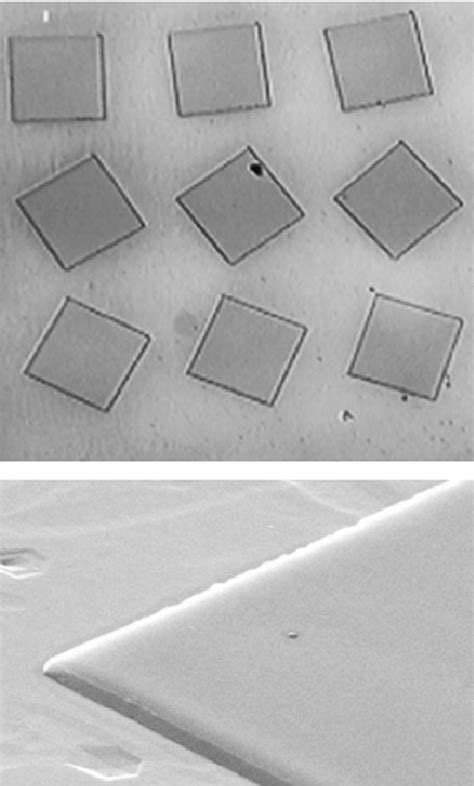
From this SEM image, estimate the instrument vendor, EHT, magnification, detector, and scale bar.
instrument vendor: JEOL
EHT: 25 kV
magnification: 2 K X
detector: SEI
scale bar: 10000 nm